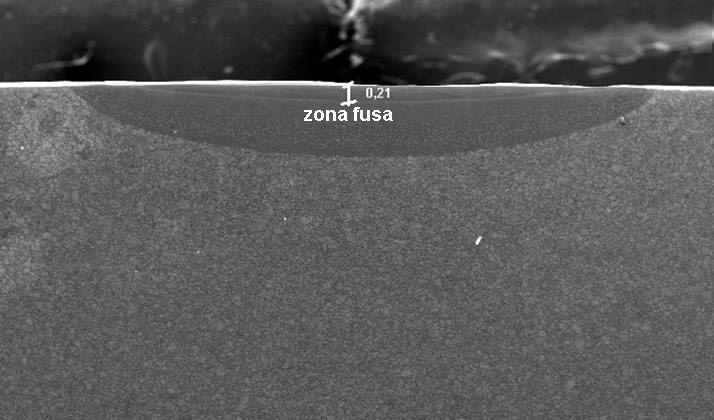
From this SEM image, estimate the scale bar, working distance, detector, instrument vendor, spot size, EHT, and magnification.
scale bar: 1000000 nm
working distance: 21 mm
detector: SE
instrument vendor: FEI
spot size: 4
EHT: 20 kV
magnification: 0.025 K X